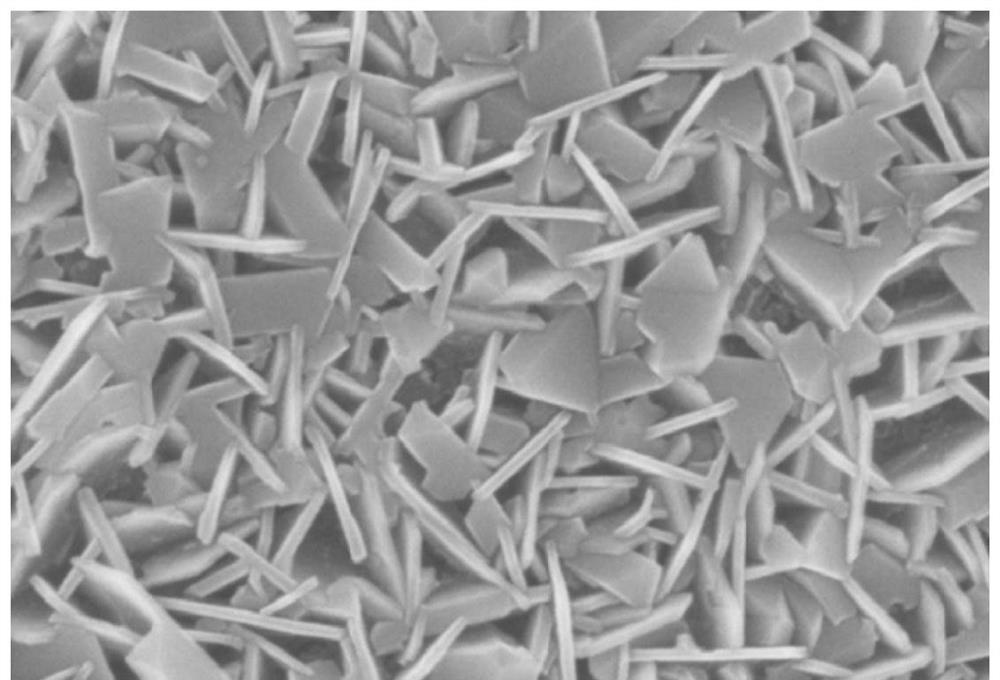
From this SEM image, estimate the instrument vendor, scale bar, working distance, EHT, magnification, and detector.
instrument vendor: Zeiss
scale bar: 200 nm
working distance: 7.4 mm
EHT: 5 kV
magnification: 50 K X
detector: InLens